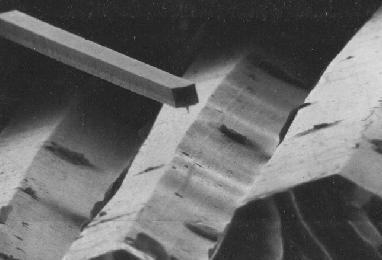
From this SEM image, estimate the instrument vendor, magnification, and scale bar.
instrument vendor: JEOL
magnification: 0.598 K X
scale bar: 50100 nm